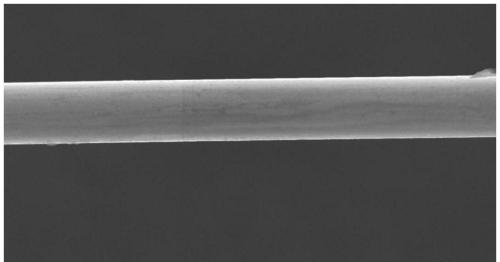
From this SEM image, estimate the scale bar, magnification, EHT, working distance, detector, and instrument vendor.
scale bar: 100000 nm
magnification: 0.5 K X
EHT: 30 kV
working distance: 9.6 mm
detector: SE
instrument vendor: Hitachi